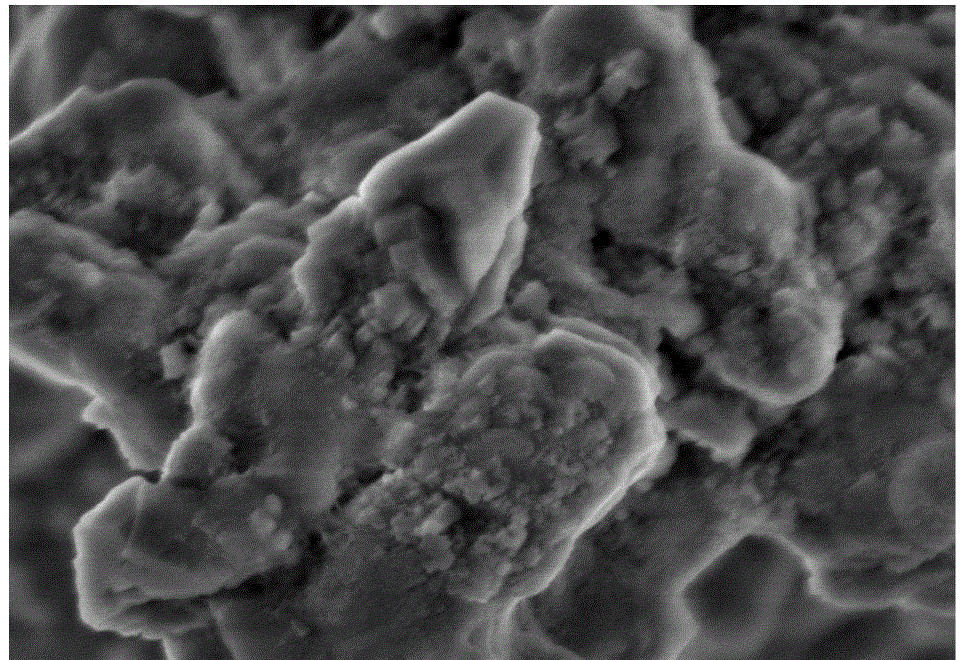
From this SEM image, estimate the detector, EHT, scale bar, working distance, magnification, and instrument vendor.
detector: SE(M)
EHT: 3 kV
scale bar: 1000 nm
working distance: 8.7 mm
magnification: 50 K X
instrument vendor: Hitachi